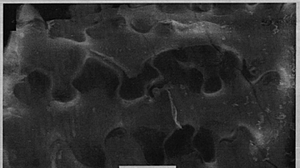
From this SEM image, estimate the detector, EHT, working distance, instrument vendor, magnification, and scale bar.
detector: SE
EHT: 20 kV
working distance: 7.6 mm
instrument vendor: FEI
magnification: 5 K X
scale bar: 10000 nm